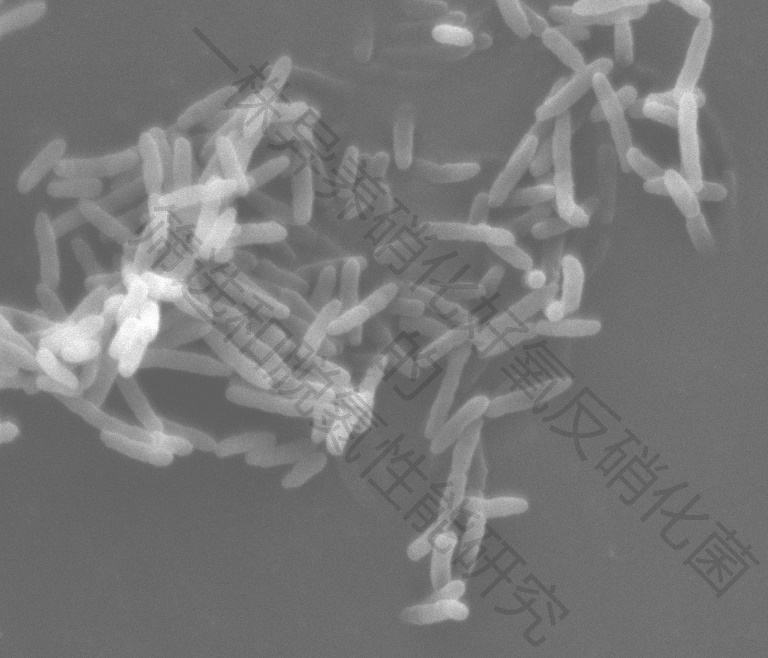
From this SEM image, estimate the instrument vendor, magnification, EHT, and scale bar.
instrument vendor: FEI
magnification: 20 K X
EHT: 15 kV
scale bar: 5000 nm